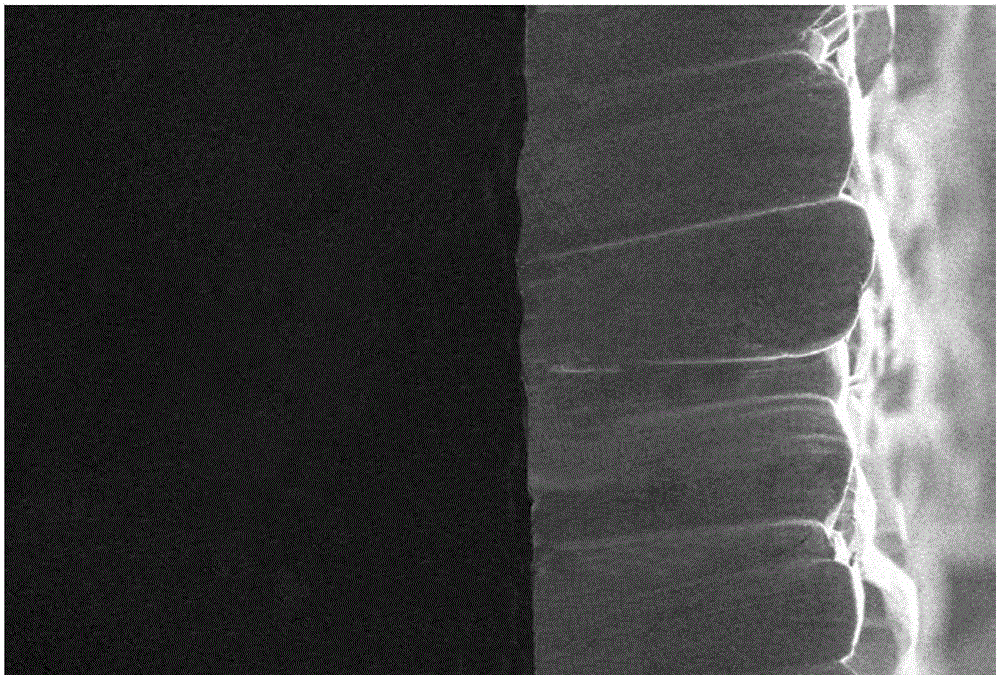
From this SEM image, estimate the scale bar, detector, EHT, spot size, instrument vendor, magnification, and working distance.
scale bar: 50000 nm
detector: SEI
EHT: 10 kV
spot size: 40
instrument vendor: JEOL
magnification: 0.3 K X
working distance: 11 mm